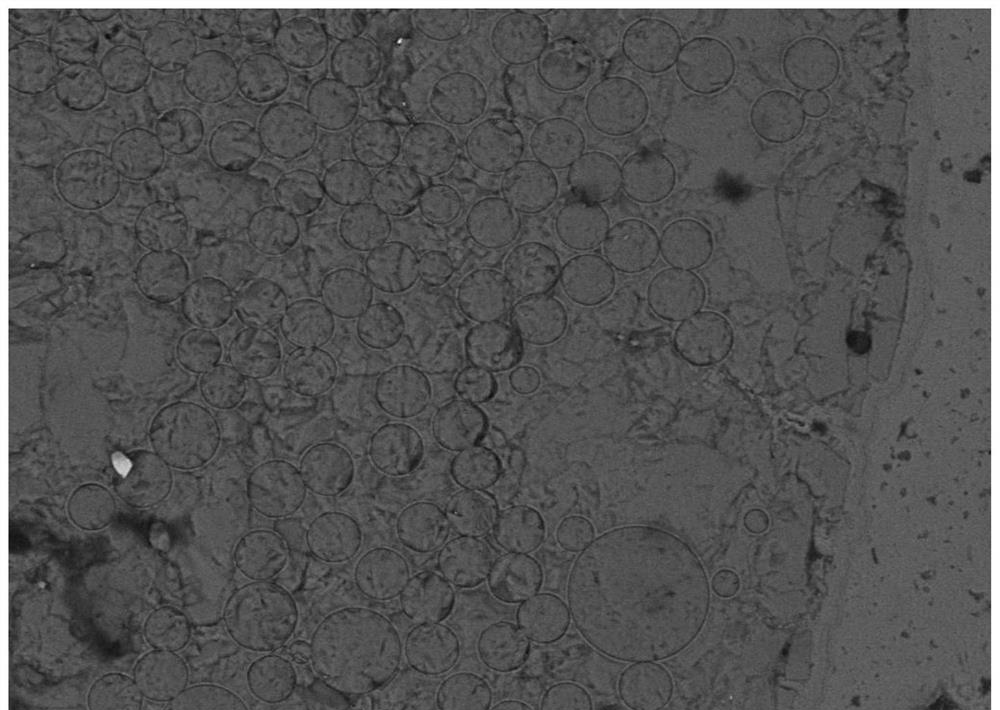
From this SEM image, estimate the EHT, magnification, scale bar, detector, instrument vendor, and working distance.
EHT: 15 kV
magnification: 0.5 K X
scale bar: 10000 nm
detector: AsB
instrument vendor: Zeiss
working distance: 11 mm